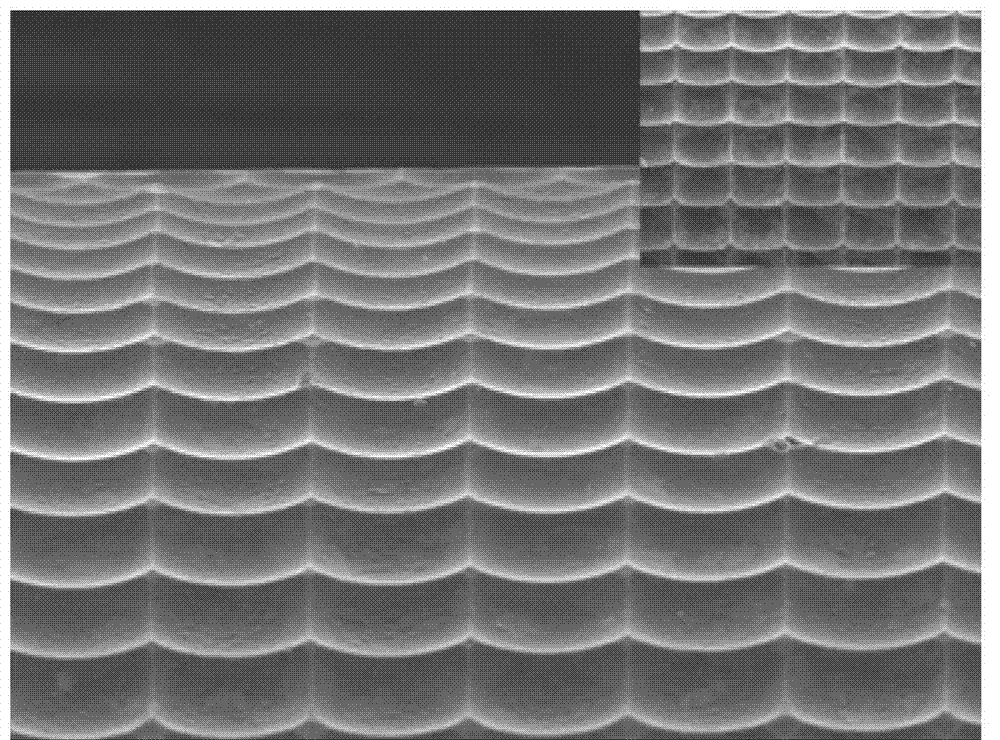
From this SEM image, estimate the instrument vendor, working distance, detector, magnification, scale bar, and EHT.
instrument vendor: JEOL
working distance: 15 mm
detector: SEI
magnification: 0.3 K X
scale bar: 10000 nm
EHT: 20 kV